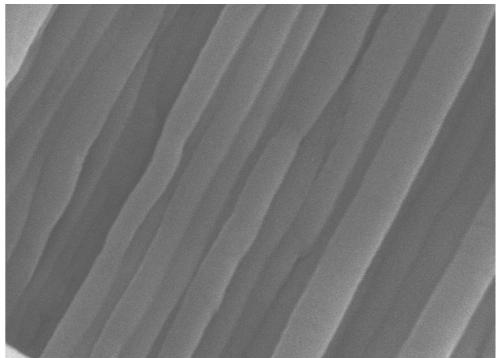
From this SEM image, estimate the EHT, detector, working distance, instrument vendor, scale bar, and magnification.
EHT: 10 kV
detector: SEI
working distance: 10 mm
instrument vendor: JEOL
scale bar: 1000 nm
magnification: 20 K X